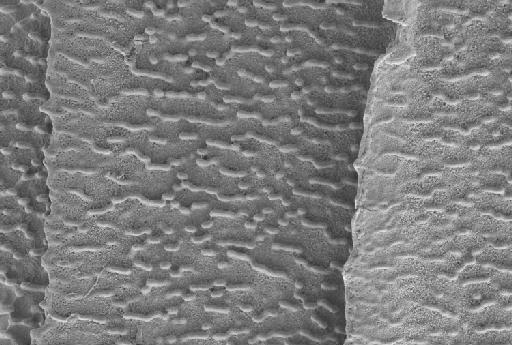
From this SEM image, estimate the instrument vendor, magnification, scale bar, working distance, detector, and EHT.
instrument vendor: Zeiss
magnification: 4.36 K X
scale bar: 10000 nm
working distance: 6.2 mm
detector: SE2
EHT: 5 kV